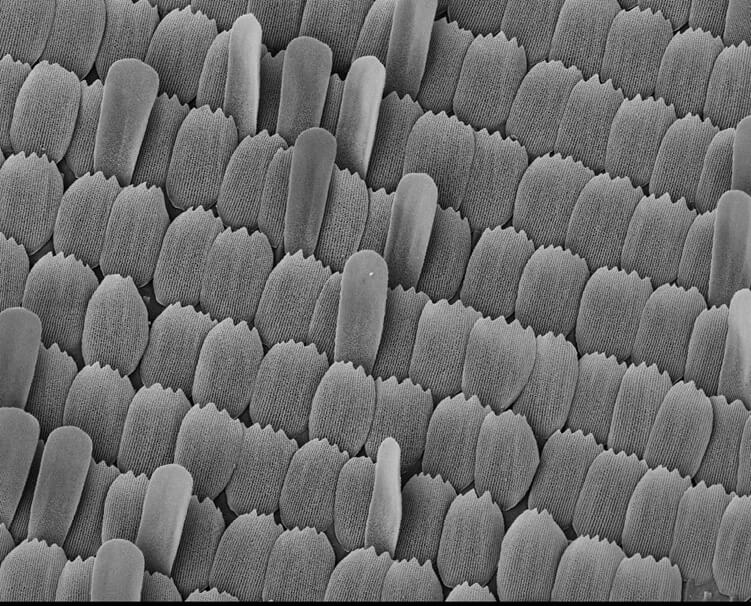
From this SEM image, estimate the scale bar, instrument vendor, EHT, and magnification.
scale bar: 100000 nm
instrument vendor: JEOL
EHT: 10 kV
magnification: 0.2 K X